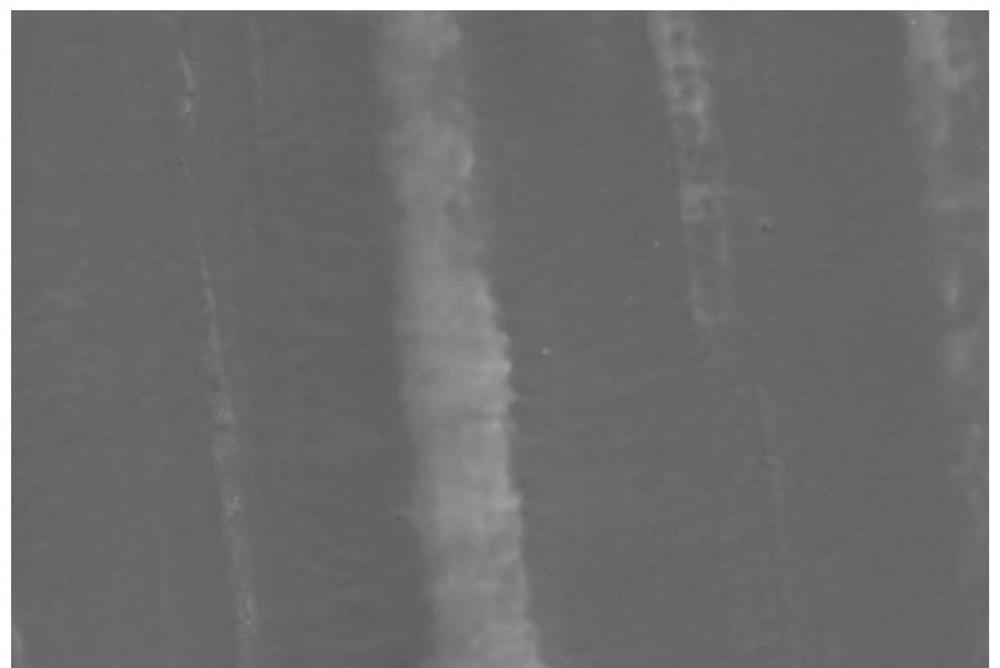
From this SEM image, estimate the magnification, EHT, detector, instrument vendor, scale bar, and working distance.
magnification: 1 K X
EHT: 10 kV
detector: SE1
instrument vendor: Zeiss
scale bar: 10000 nm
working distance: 8.5 mm